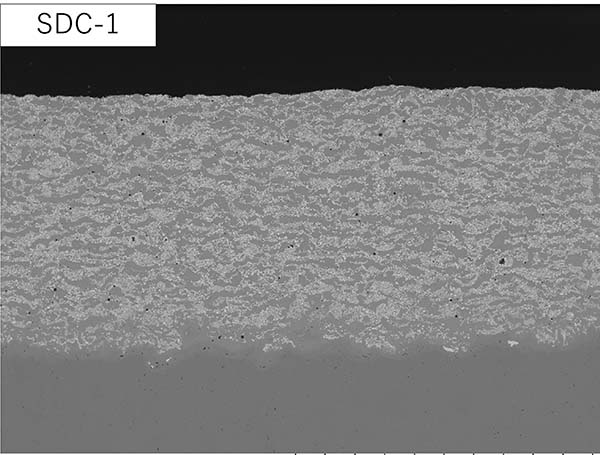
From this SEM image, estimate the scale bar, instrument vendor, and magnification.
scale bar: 1000000 nm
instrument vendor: Hitachi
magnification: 0.08 K X